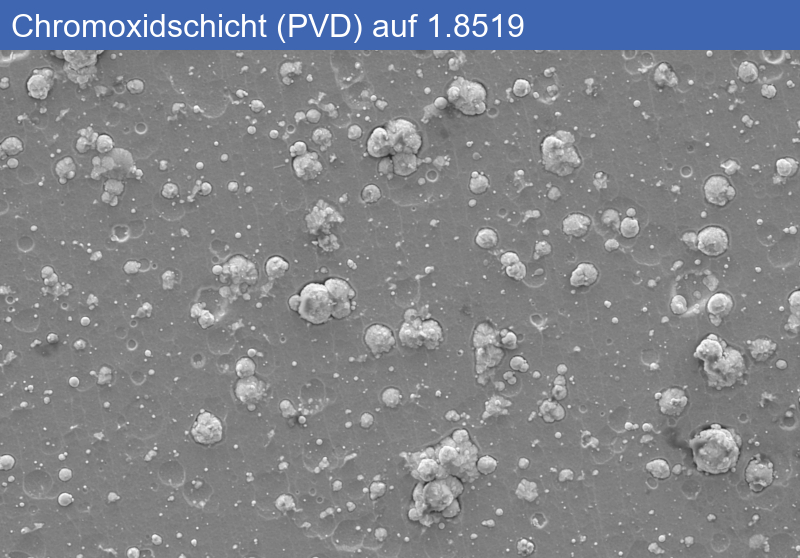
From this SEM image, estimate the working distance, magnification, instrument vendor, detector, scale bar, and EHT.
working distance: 11.4 mm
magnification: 2 K X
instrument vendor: Zeiss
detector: SE2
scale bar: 2000 nm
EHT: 5 kV